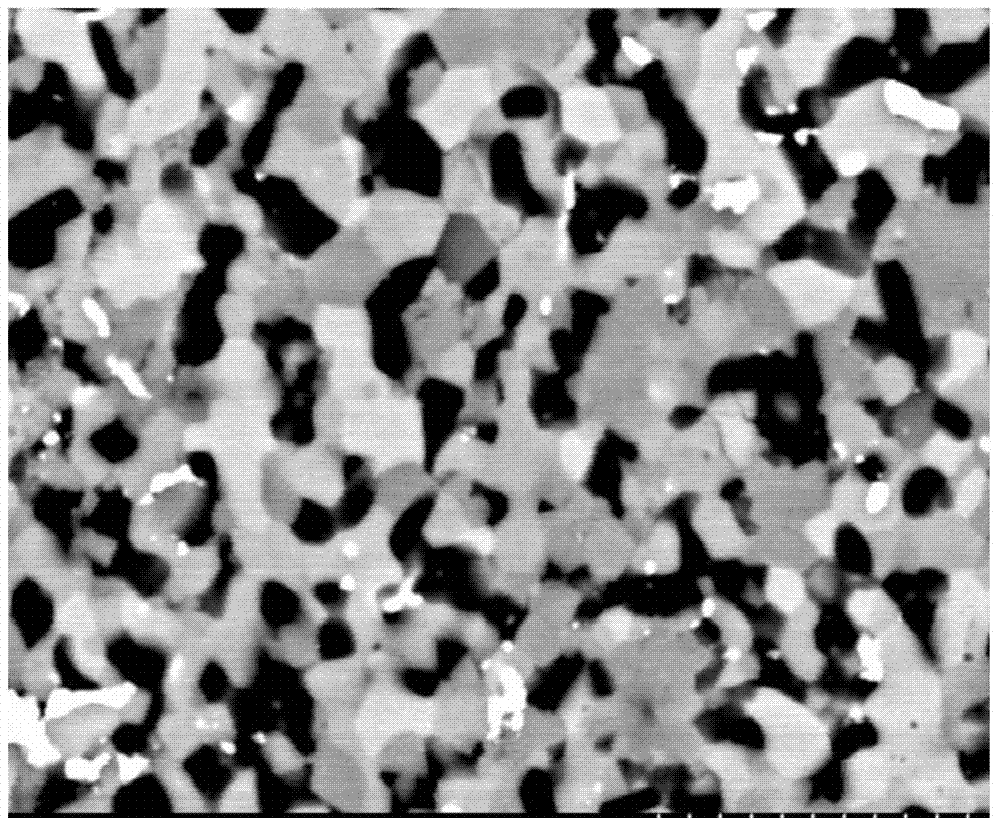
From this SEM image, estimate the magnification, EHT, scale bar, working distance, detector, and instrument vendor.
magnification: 8 K X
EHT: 15 kV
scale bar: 5000 nm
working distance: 11.9 mm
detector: YAGBSE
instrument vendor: Hitachi